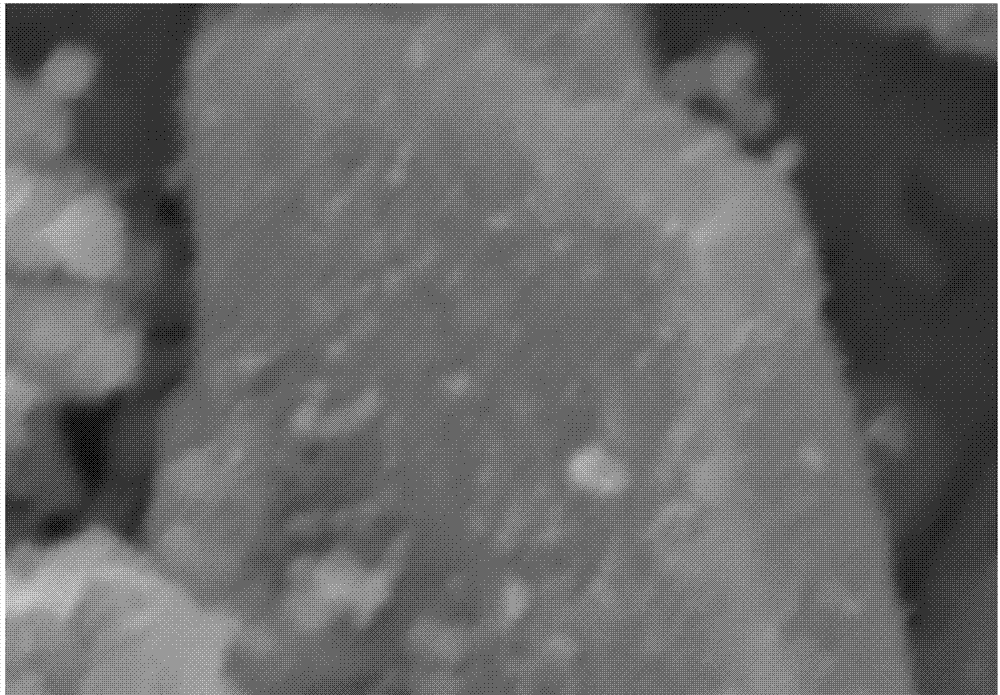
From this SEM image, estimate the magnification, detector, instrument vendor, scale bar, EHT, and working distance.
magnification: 10 K X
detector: SE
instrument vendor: Hitachi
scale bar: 5000 nm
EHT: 20.2 kV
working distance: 19.2 mm